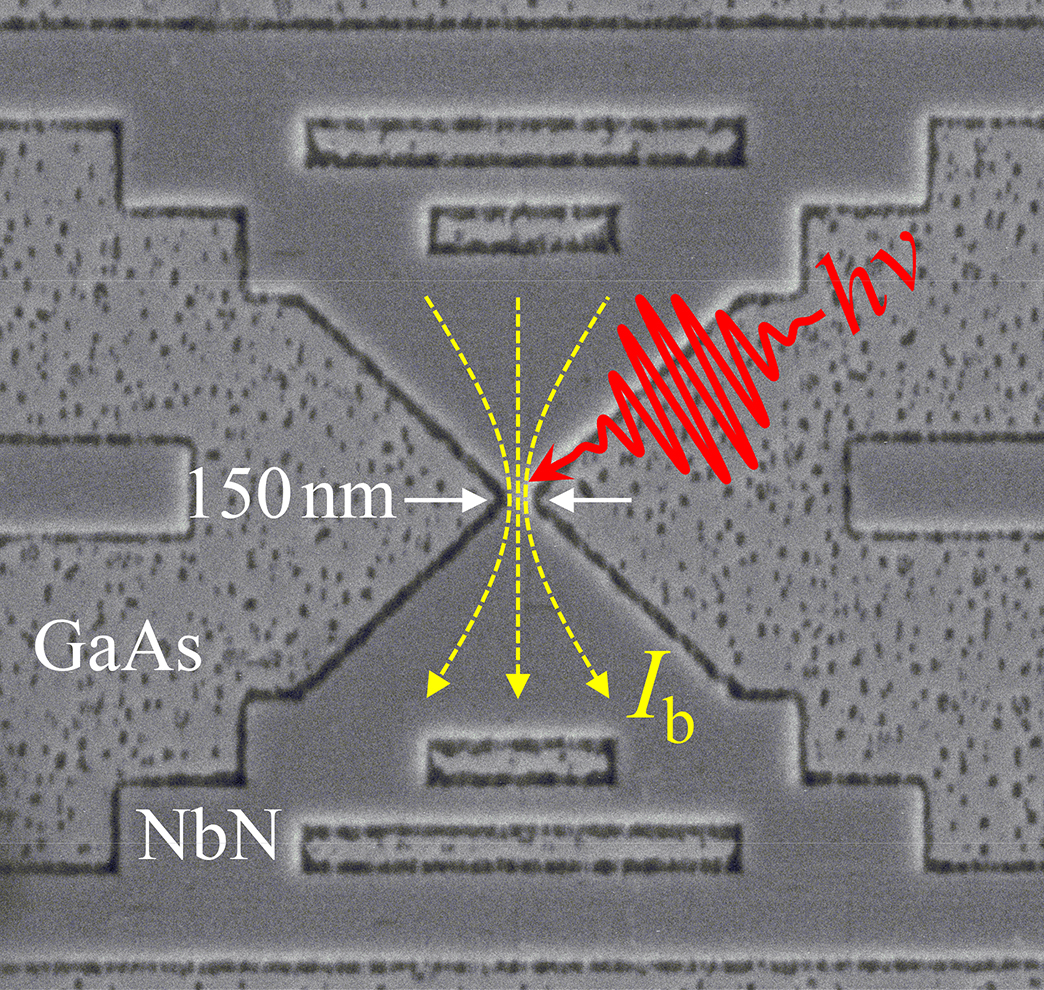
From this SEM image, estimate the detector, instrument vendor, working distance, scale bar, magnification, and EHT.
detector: LEI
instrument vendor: JEOL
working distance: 15.4 mm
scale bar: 1000 nm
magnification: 15 K X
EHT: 5 kV